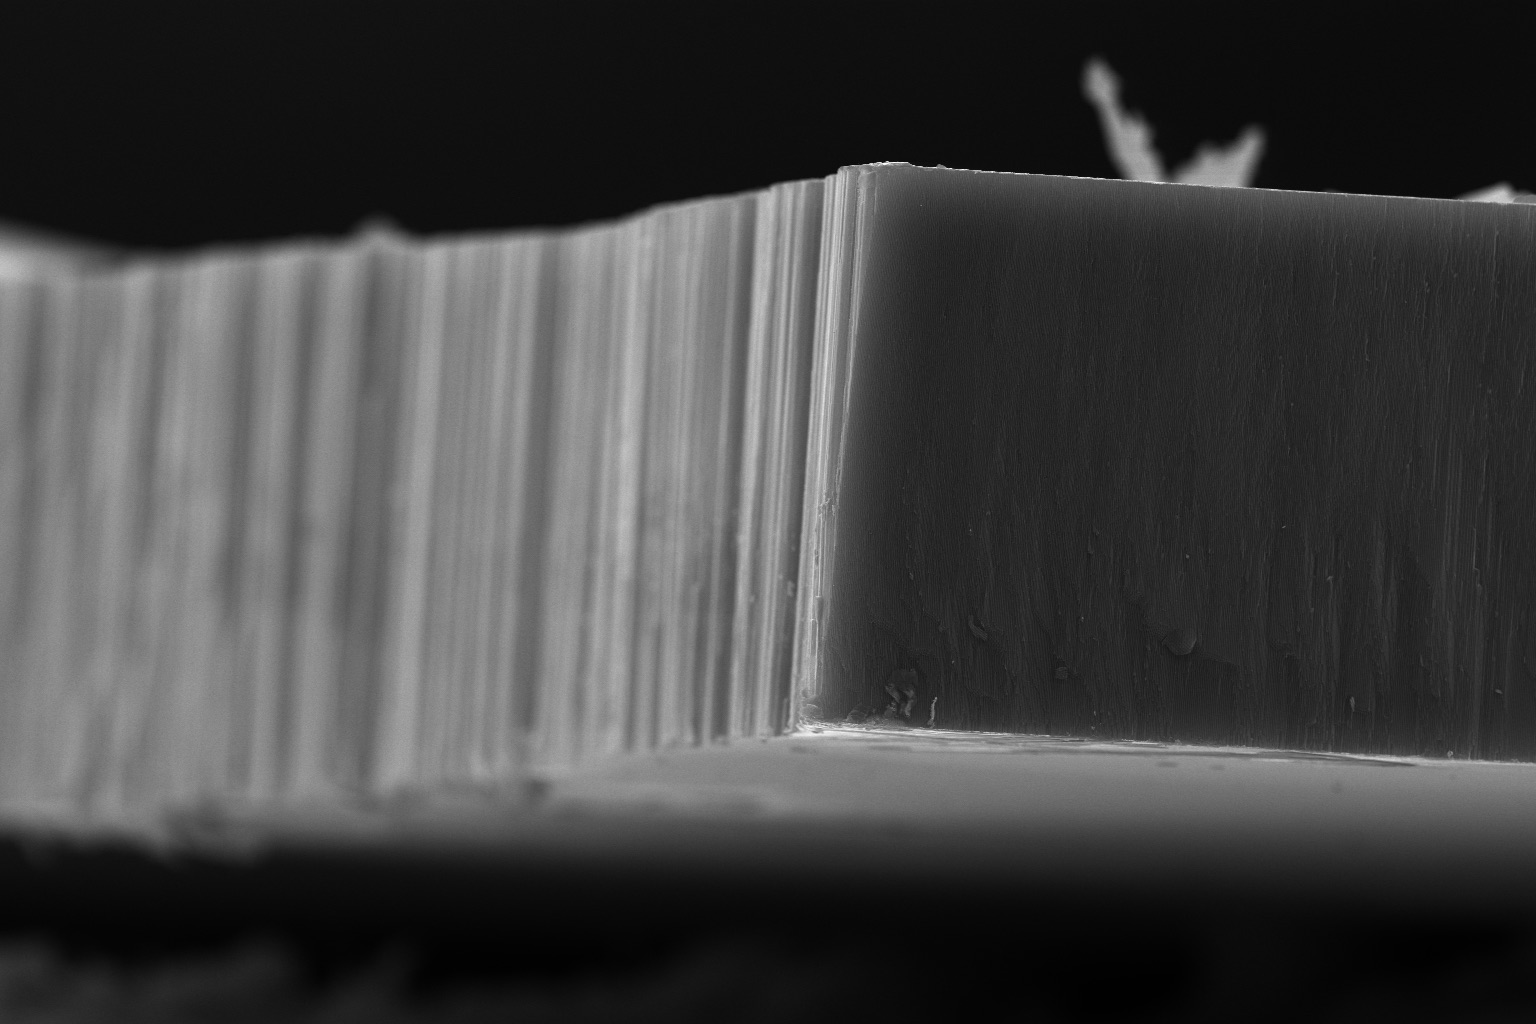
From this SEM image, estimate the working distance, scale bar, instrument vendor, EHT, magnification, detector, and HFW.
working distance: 4.9 mm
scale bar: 20000 nm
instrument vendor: FEI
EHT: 20 kV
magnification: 5 K X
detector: ETD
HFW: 82.9 µm